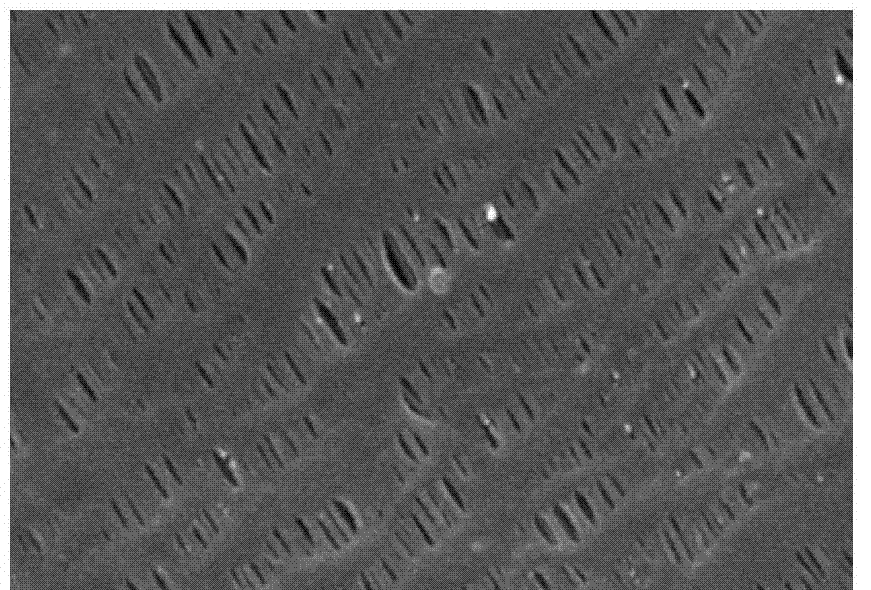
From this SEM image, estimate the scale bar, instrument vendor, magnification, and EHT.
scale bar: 1000 nm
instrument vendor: JEOL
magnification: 20 K X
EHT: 15 kV